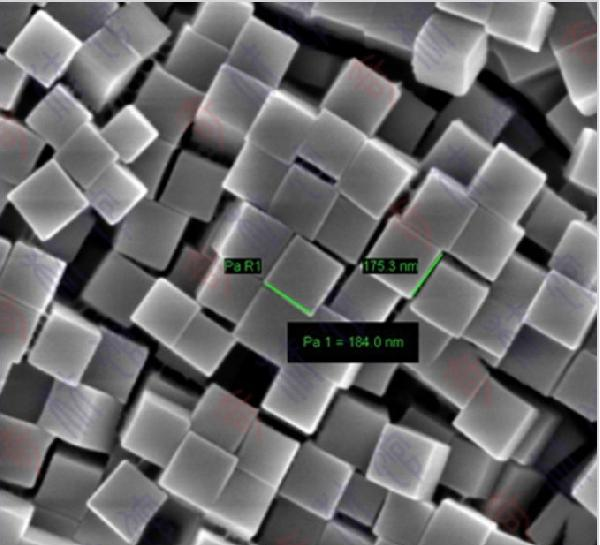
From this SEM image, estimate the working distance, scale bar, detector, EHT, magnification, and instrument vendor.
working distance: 5.7 mm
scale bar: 200 nm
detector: InLens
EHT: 3 kV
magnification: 41.91 K X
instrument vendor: Zeiss